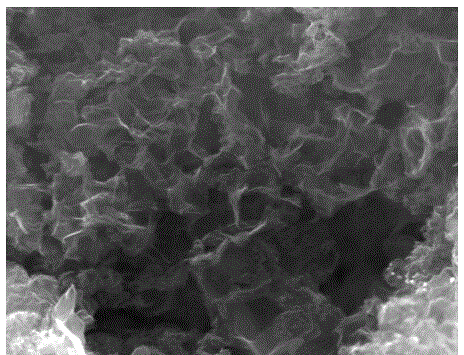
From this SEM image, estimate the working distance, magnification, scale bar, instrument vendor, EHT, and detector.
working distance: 6.6 mm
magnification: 80 K X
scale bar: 1000 nm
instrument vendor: FEI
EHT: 20 kV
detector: SE